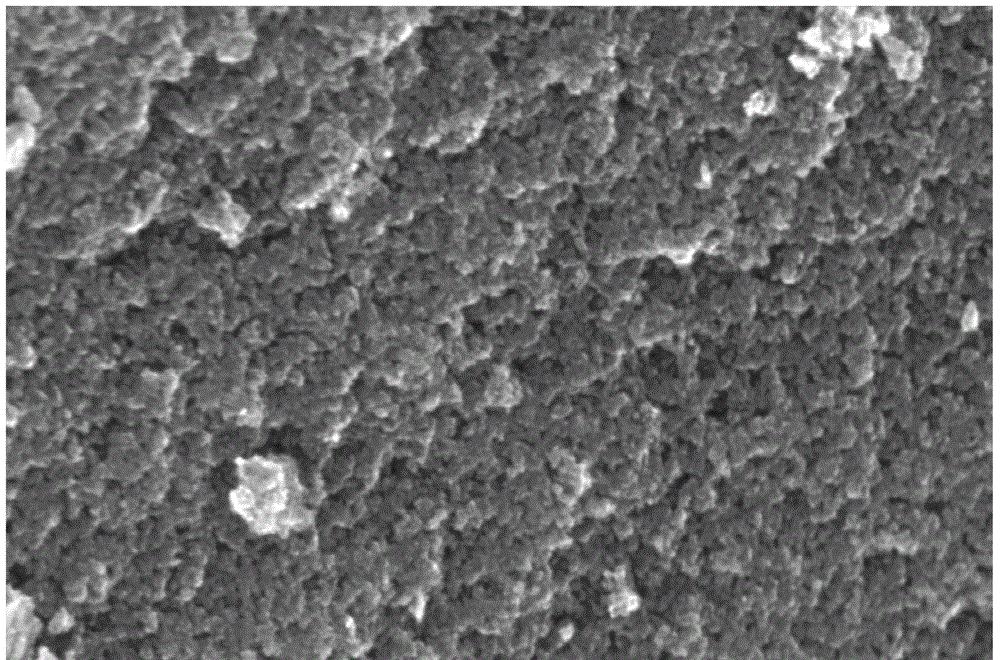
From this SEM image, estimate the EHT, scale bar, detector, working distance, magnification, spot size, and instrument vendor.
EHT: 5 kV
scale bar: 500 nm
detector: TLD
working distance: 4.9 mm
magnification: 240 K X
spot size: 3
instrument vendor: FEI